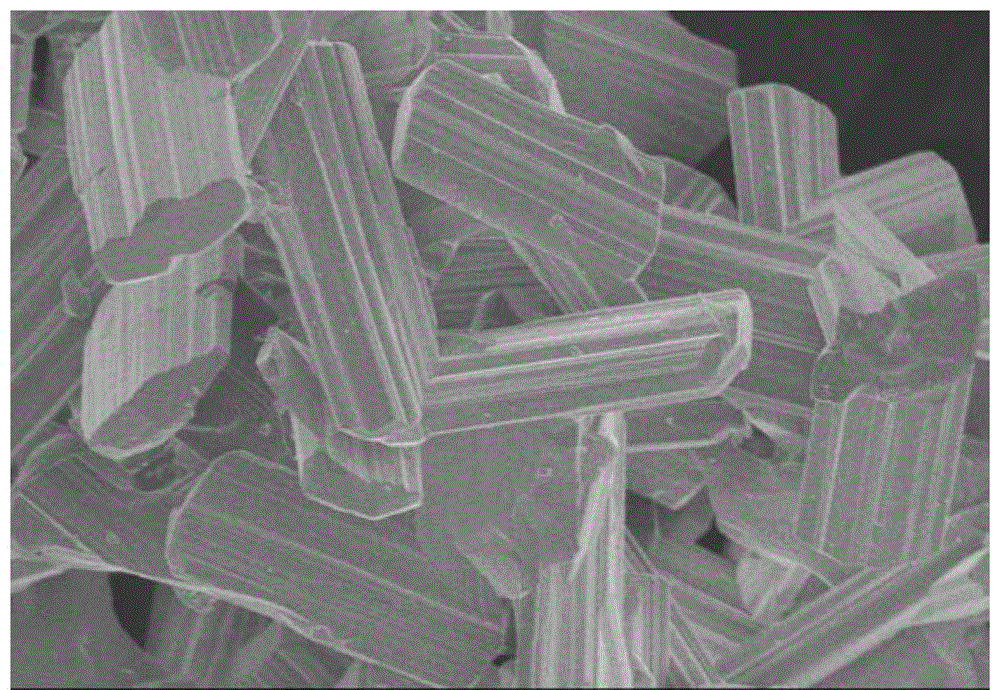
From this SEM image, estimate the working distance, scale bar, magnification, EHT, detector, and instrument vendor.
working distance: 15 mm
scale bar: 10000 nm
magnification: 1 K X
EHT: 5 kV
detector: SE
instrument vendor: Hitachi